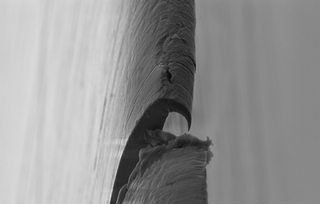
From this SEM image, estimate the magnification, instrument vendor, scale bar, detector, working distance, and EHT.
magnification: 5 K X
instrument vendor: Zeiss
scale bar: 2000 nm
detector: SE2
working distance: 6.5 mm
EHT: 5 kV